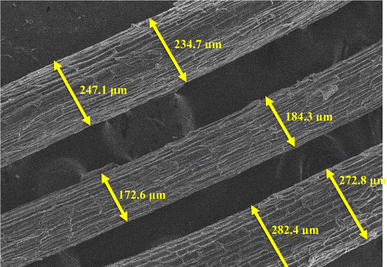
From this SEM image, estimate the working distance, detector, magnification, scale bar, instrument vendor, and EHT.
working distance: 9.7 mm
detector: SE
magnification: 0.1 K X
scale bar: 500000 nm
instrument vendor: Hitachi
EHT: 10 kV